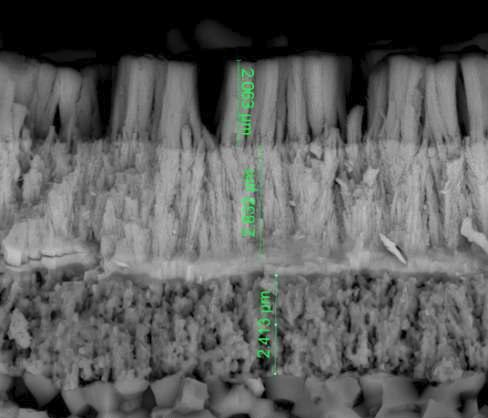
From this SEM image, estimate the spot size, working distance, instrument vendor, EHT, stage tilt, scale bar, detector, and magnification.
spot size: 3.5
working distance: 10 mm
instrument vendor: FEI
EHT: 15 kV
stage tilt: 0°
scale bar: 5000 nm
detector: vCD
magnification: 25 K X